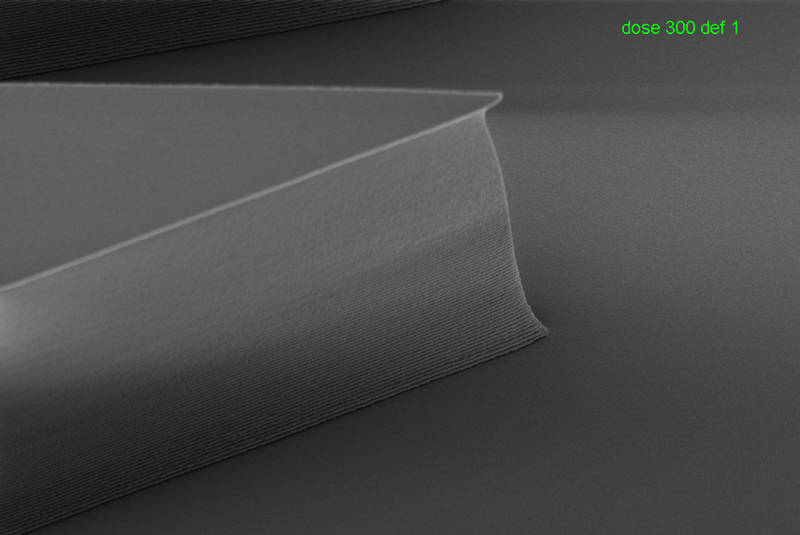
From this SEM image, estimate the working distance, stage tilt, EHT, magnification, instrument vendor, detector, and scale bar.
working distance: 4.7 mm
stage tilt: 30°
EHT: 1.5 kV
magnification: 6.32 K X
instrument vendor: Zeiss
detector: InLens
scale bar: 1000 nm